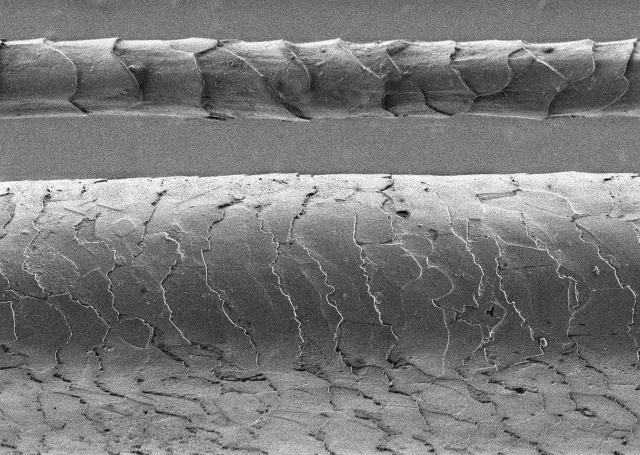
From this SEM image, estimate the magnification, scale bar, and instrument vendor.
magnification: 1 K X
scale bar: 50000 nm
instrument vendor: Hitachi